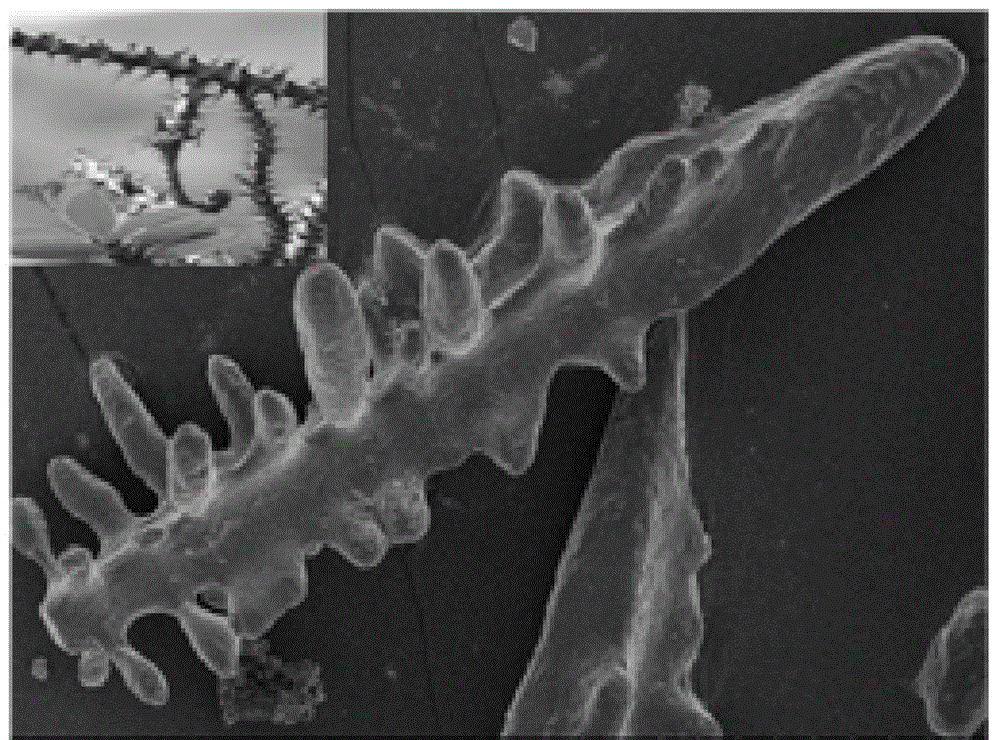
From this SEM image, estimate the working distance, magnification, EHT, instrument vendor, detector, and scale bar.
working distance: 8.4 mm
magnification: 0.5 K X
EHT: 3 kV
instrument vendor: Hitachi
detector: SE(M)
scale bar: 100000 nm